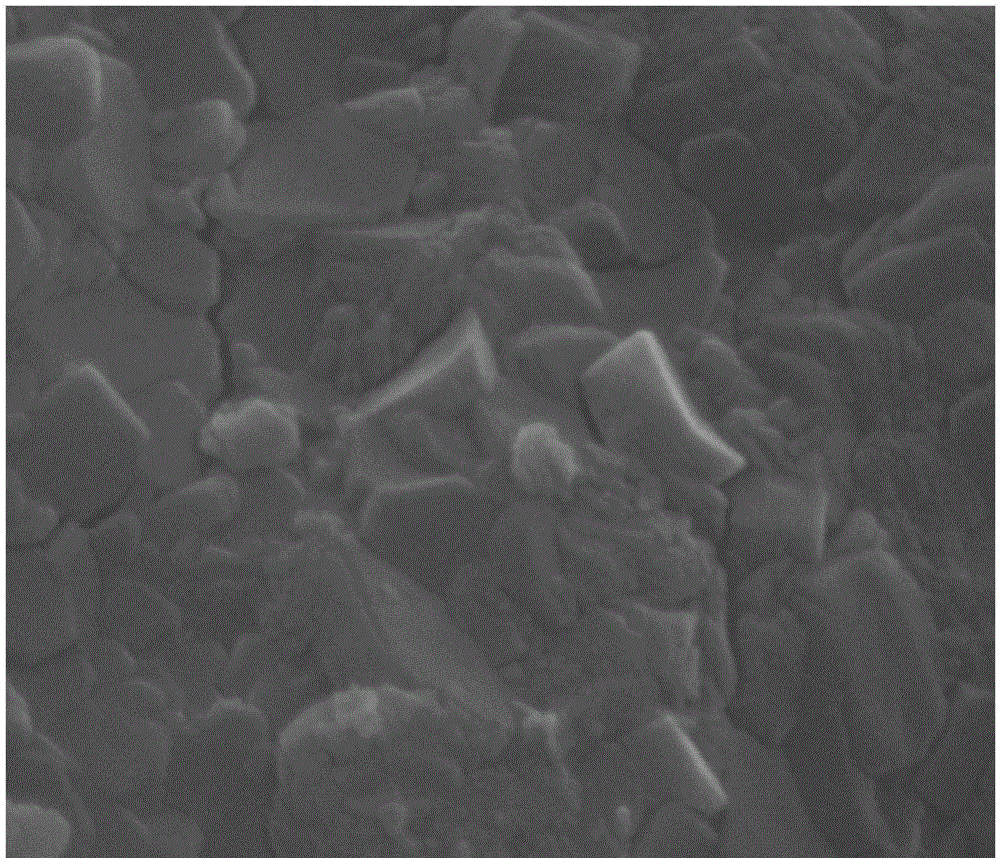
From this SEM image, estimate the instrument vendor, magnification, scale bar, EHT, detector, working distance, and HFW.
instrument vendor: FEI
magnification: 100 K X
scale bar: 300 nm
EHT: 20 kV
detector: ETD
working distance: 9.8 mm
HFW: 1.35 µm